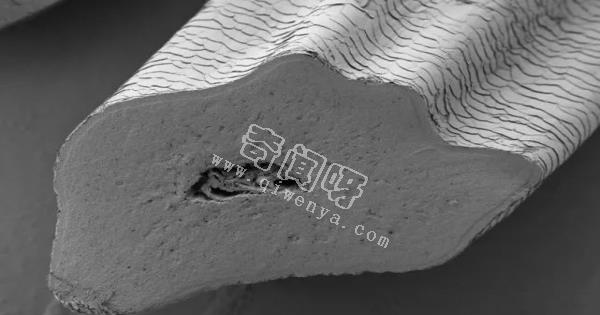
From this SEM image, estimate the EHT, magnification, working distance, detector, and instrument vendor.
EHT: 1 kV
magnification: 0.5 K X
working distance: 4.3 mm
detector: SE2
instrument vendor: Zeiss